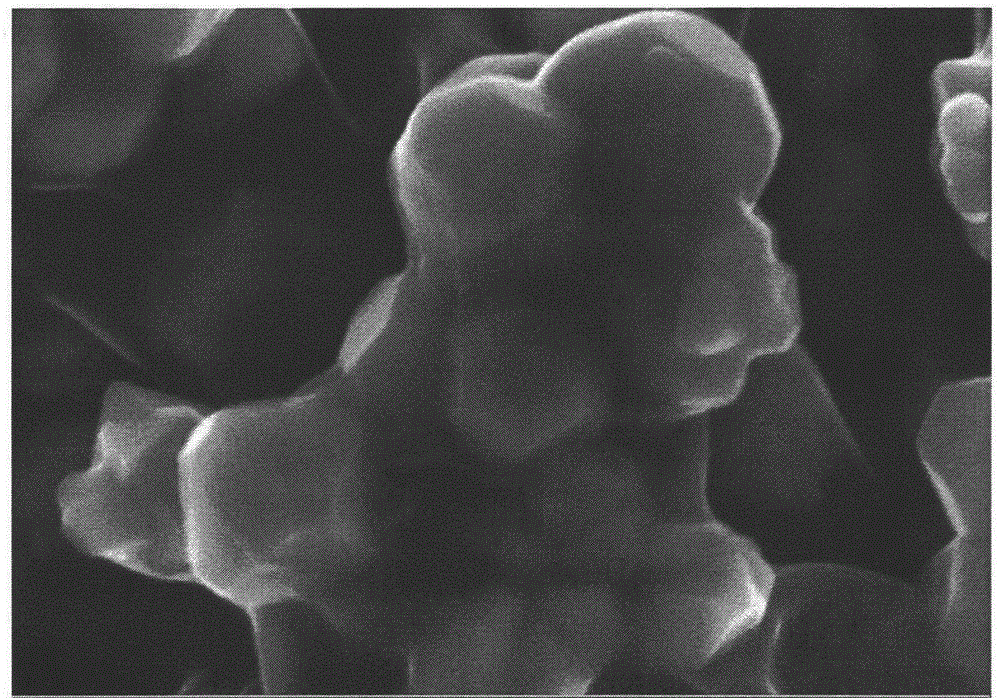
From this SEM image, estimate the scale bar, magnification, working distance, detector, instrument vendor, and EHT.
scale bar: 200 nm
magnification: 100 K X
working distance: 6.5 mm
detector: InLens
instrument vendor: Zeiss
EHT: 5 kV